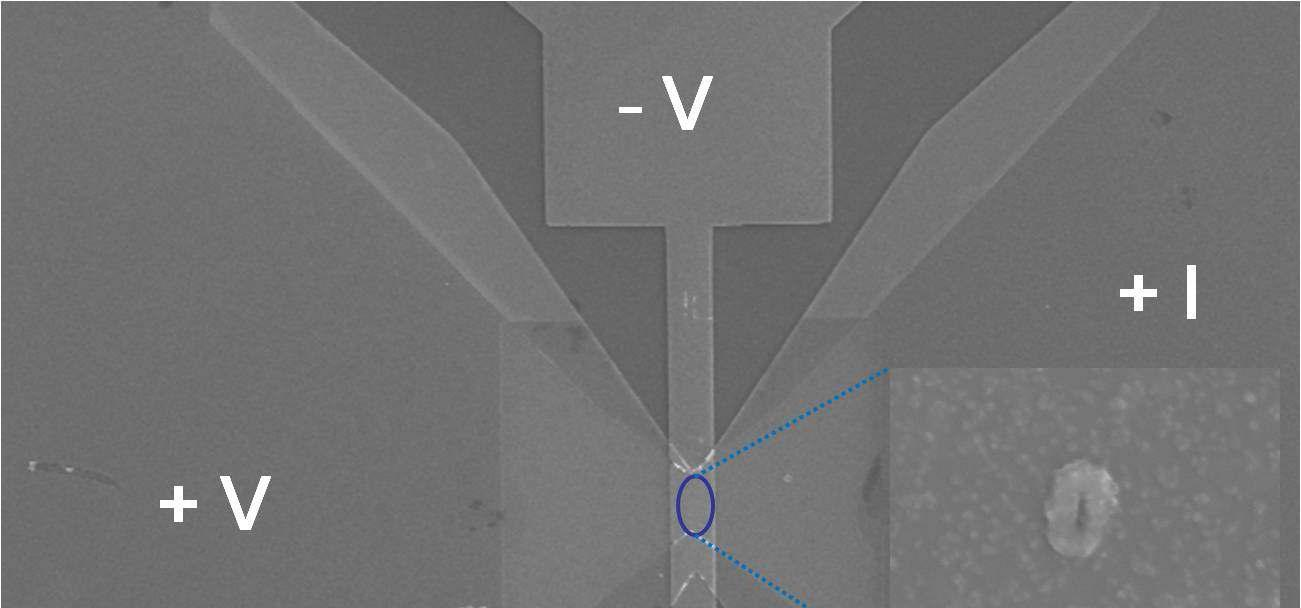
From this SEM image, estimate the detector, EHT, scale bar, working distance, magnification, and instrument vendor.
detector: SE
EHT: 15 kV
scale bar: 100000 nm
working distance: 15 mm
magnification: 0.45 K X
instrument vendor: Hitachi